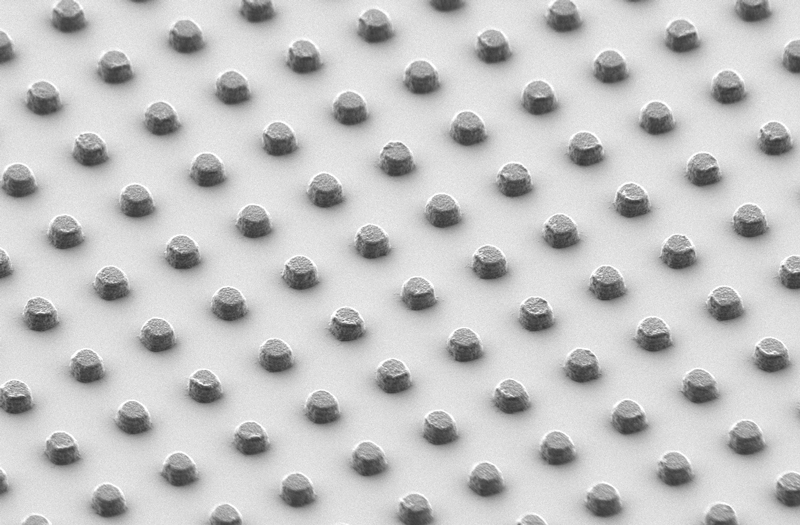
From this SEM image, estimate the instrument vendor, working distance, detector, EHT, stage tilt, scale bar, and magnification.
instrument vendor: Zeiss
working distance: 6.6 mm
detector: HE-SE2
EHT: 1 kV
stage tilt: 45.4°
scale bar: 1000 nm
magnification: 4.99 K X